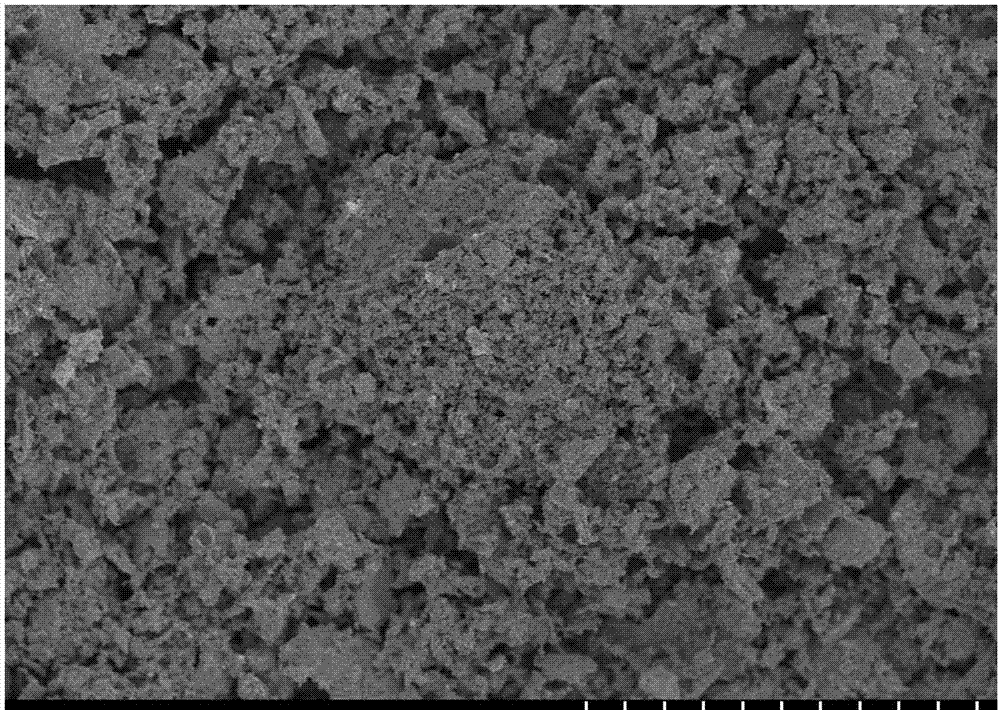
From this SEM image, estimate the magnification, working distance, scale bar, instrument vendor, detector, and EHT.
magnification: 1 K X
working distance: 8 mm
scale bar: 50000 nm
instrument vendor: Hitachi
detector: SE(M)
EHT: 3 kV